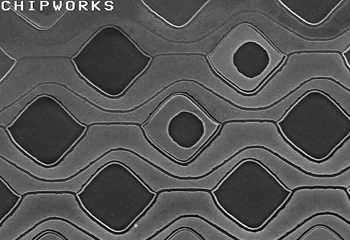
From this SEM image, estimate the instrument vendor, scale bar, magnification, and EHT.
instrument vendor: JEOL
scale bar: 3000 nm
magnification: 10 K X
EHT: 5 kV